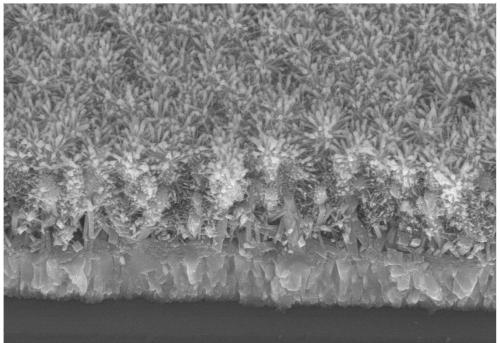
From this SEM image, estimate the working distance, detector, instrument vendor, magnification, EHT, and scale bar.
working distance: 12 mm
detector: SE(M)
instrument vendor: Hitachi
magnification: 20 K X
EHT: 10 kV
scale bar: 2000 nm